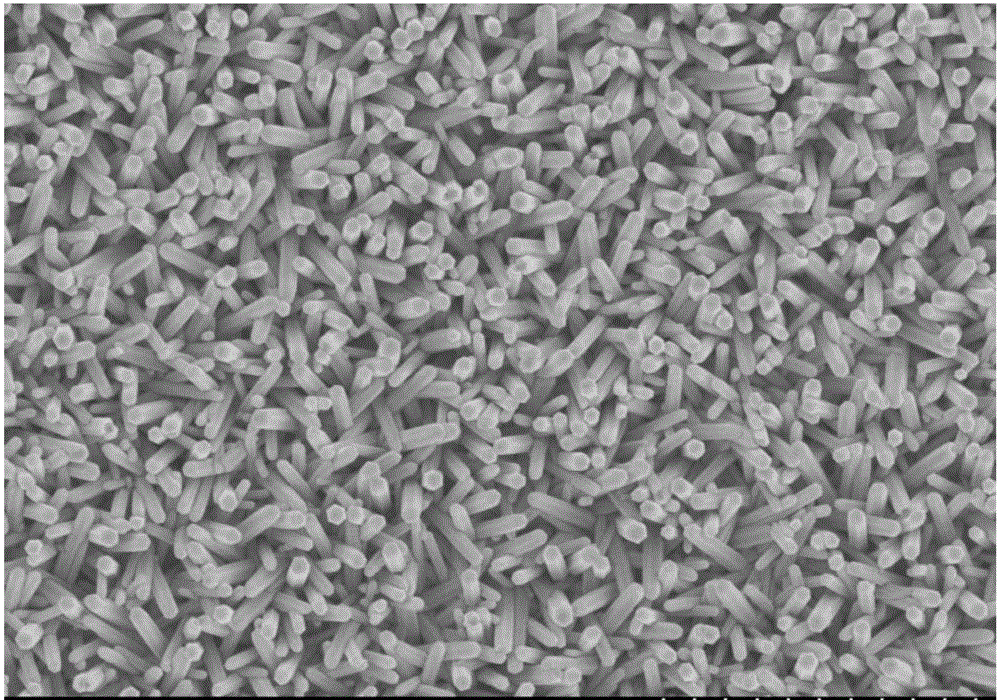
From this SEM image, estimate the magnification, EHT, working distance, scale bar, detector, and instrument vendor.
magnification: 20 K X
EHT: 3 kV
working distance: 9.5 mm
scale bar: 2000 nm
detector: SE(M)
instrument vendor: Hitachi